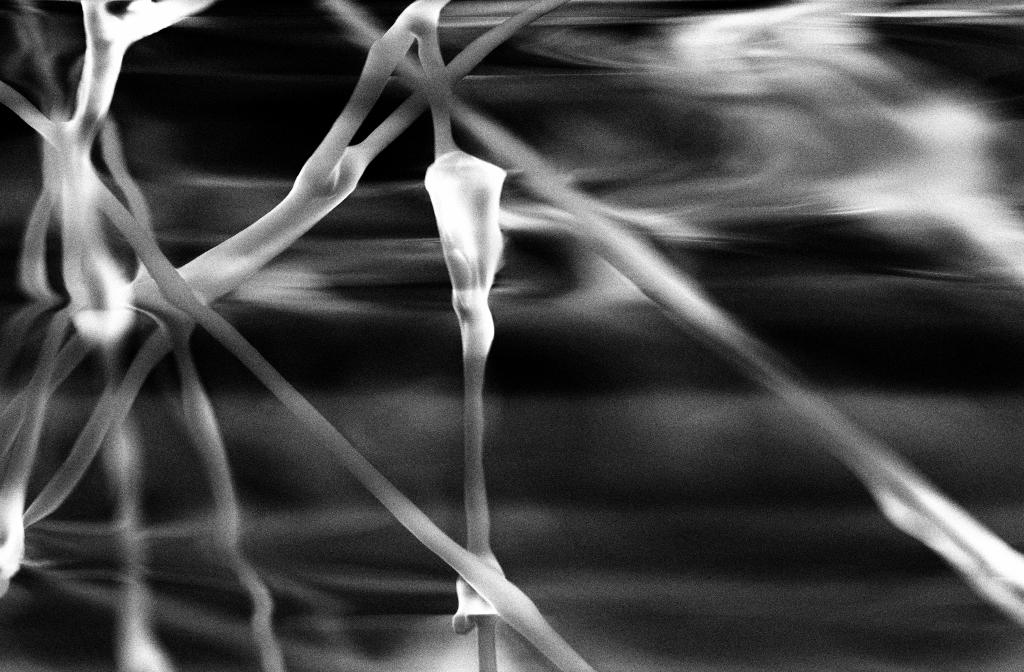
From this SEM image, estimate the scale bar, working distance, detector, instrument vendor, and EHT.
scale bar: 20000 nm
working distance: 17 mm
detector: SE2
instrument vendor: Zeiss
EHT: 20 kV